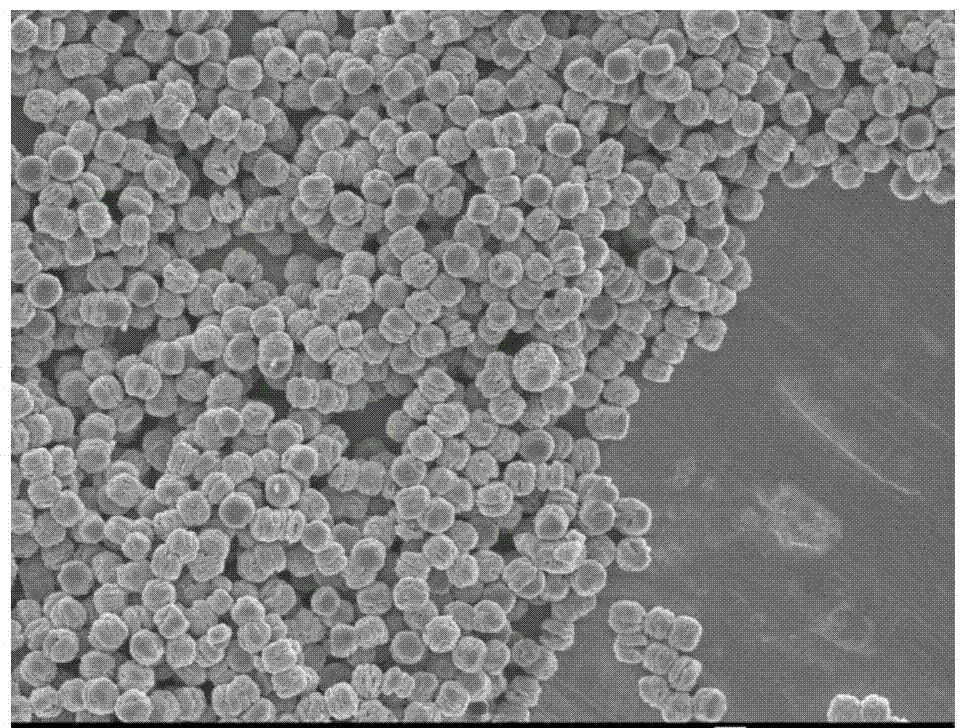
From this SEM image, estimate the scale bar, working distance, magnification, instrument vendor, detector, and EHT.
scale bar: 1000 nm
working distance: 7.8 mm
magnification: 4 K X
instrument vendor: JEOL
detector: SEI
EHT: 5 kV